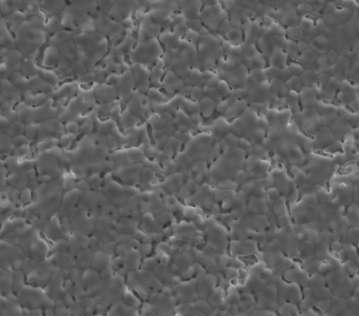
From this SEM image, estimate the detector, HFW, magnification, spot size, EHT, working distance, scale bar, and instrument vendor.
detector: ETD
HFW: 59.7 µm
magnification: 5 K X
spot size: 4.5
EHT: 15 kV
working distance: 10.7 mm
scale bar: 20000 nm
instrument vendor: FEI